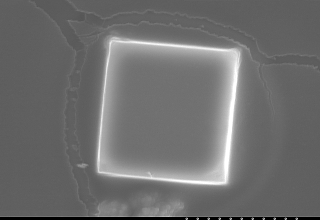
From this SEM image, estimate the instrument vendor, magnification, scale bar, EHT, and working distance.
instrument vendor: JEOL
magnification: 4.5 K X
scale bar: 10000 nm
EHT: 15 kV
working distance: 15 mm